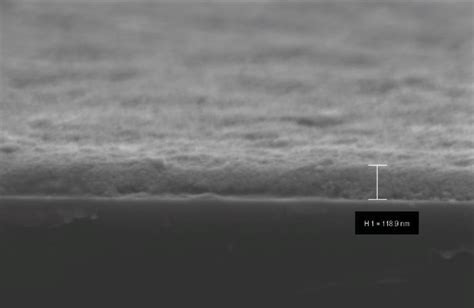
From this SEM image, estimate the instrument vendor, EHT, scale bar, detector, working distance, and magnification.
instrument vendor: Zeiss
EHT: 3 kV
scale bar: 200 nm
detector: InLens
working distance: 2.6 mm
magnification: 69.48 K X